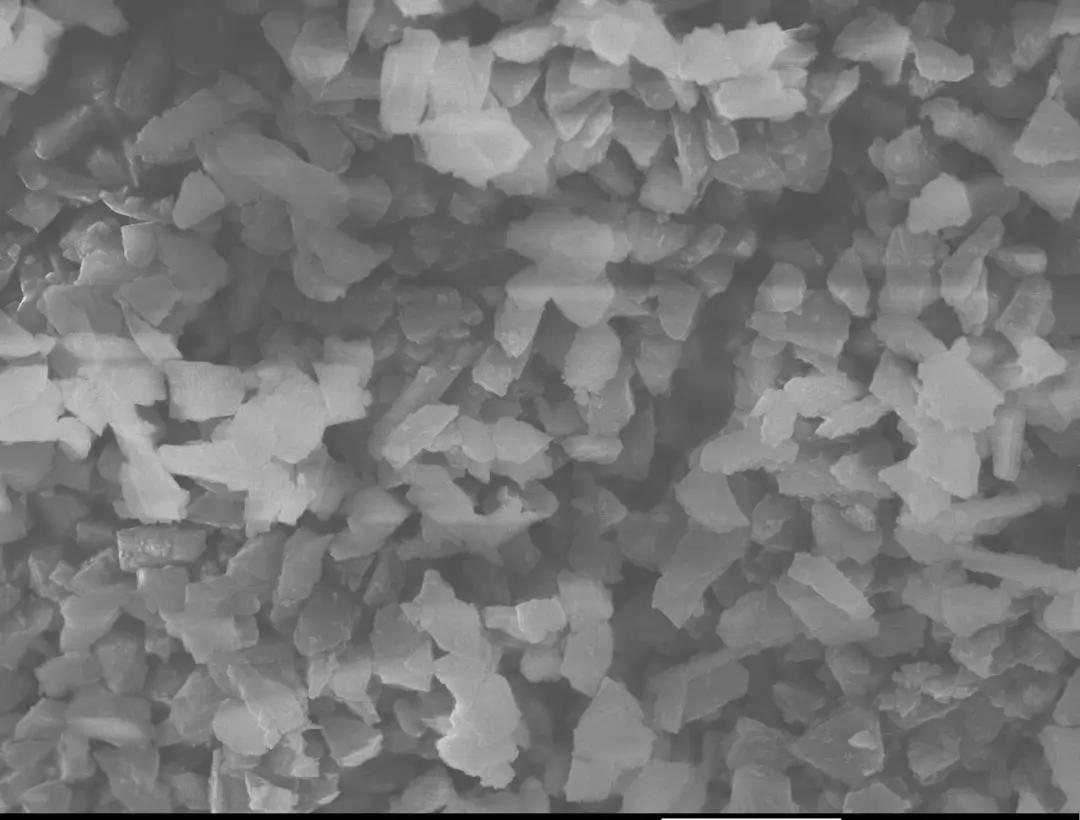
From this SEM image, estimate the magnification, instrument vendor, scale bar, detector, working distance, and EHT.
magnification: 20 K X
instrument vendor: JEOL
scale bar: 1000 nm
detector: SEI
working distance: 5.9 mm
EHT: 6 kV